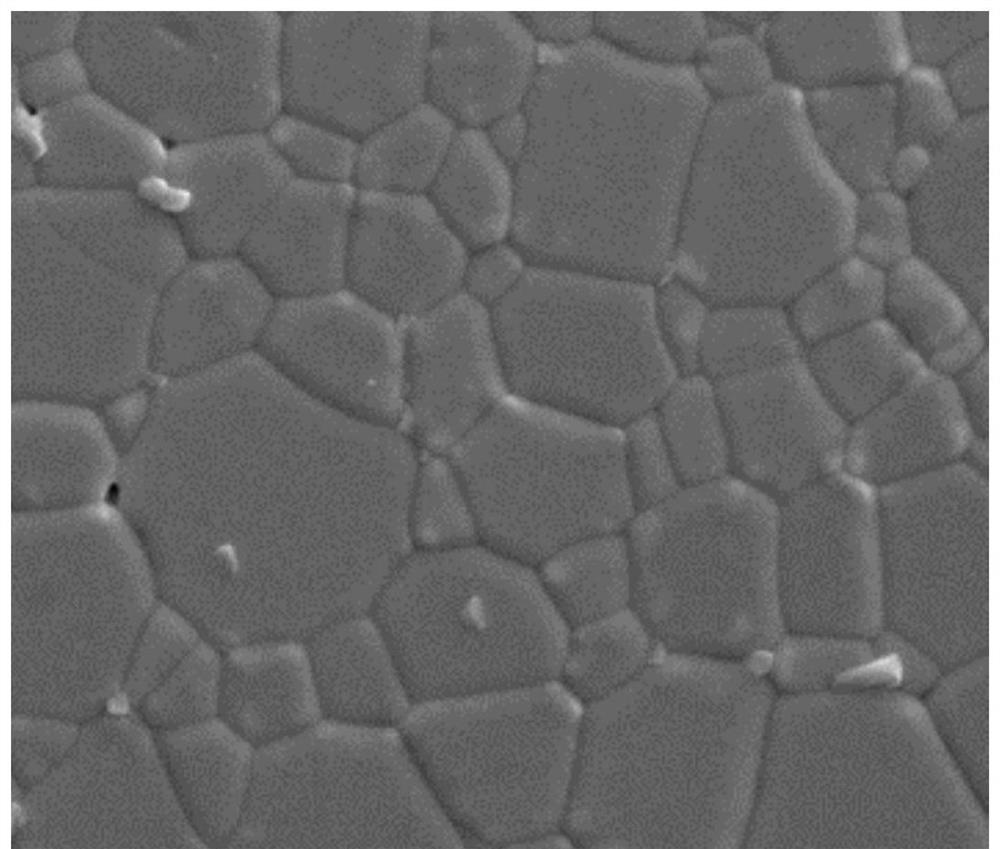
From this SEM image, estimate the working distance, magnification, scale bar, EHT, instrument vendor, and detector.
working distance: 5.8 mm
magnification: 20 K X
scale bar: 4000 nm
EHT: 10 kV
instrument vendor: FEI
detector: ETD